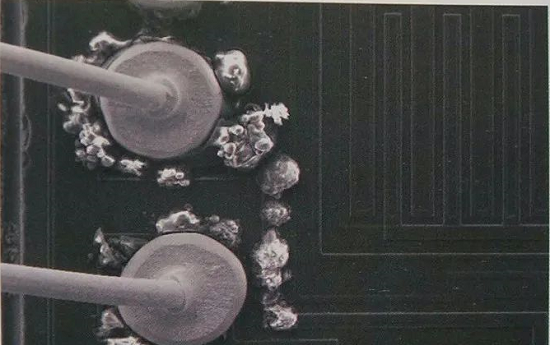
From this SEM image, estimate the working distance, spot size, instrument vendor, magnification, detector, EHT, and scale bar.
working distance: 10 mm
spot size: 3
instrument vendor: FEI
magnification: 0.2 K X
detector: SE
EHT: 15 kV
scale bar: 100000 nm